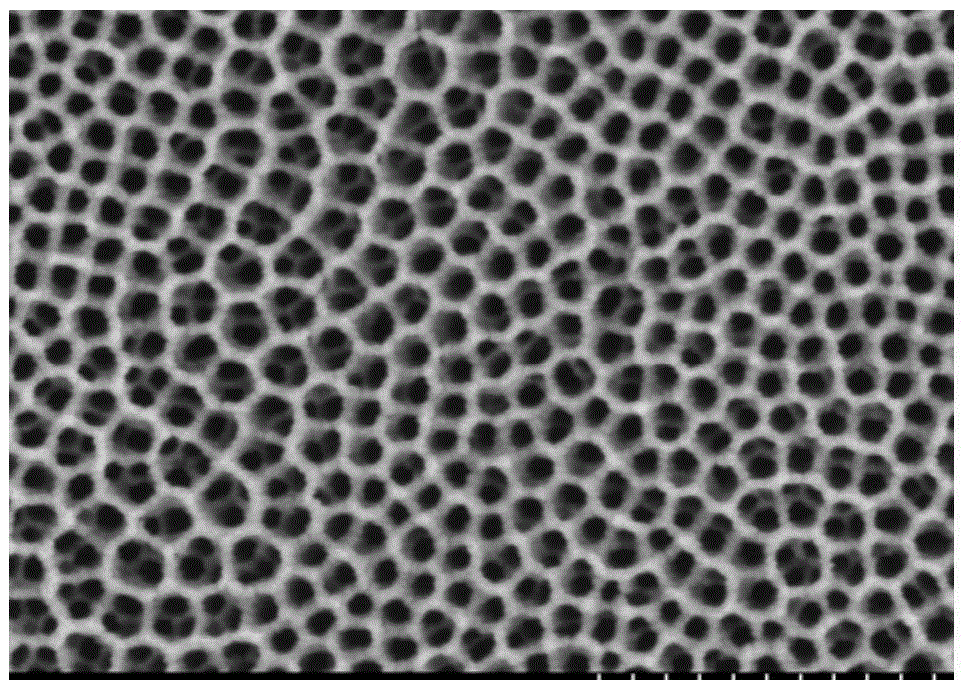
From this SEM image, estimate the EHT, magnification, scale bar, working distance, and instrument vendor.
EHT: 3 kV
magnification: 45 K X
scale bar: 1000 nm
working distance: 17.2 mm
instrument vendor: Hitachi